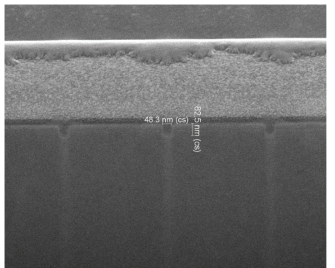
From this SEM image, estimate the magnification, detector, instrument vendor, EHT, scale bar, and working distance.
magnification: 75 K X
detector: TLD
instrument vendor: FEI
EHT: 5 kV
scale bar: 500 nm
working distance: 4.1 mm